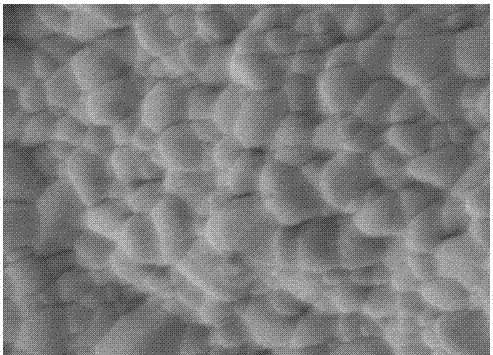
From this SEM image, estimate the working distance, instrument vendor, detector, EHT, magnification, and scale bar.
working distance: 9.7 mm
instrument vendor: JEOL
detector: LEI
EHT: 5 kV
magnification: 10 K X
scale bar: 1000 nm